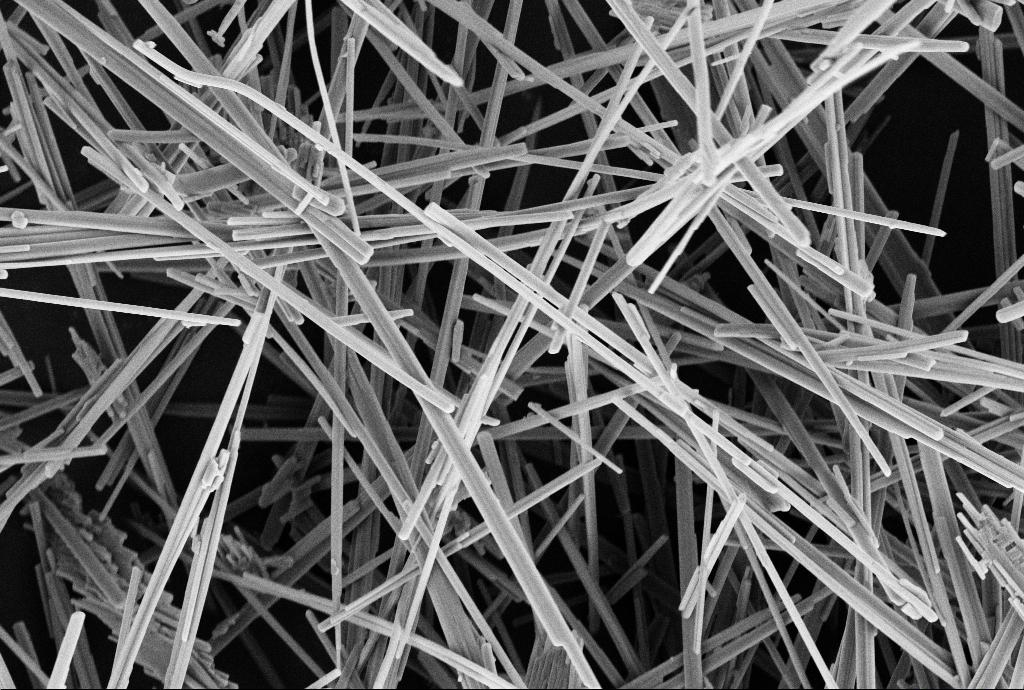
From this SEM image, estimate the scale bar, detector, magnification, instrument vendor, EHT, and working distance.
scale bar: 3000 nm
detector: SE2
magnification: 5 K X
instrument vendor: Zeiss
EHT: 4 kV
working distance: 9.9 mm